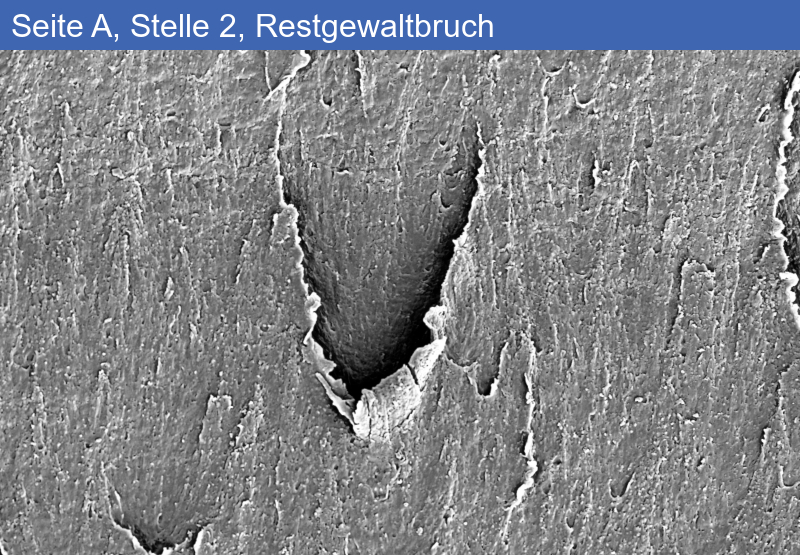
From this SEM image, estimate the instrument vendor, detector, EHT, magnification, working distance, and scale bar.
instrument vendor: Zeiss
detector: SE2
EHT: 5 kV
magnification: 1 K X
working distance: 9 mm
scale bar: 10000 nm